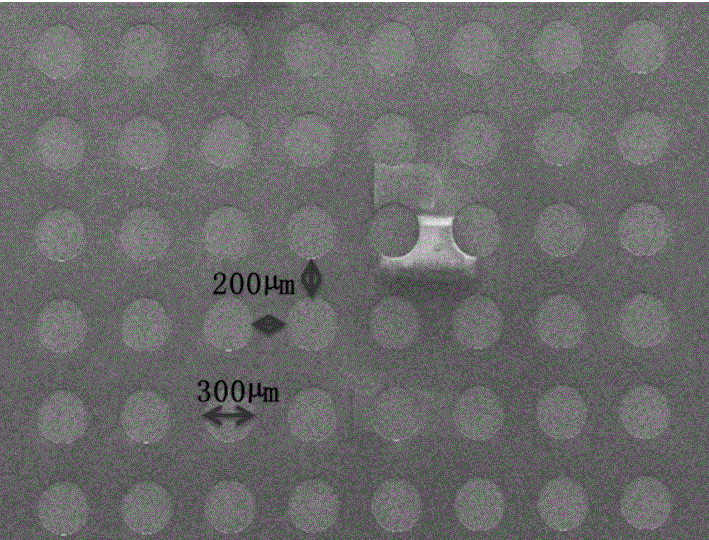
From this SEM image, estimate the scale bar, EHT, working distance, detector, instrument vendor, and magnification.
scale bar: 1000000 nm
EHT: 5 kV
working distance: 11.1 mm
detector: SE(M)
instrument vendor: Hitachi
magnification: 0.03 K X